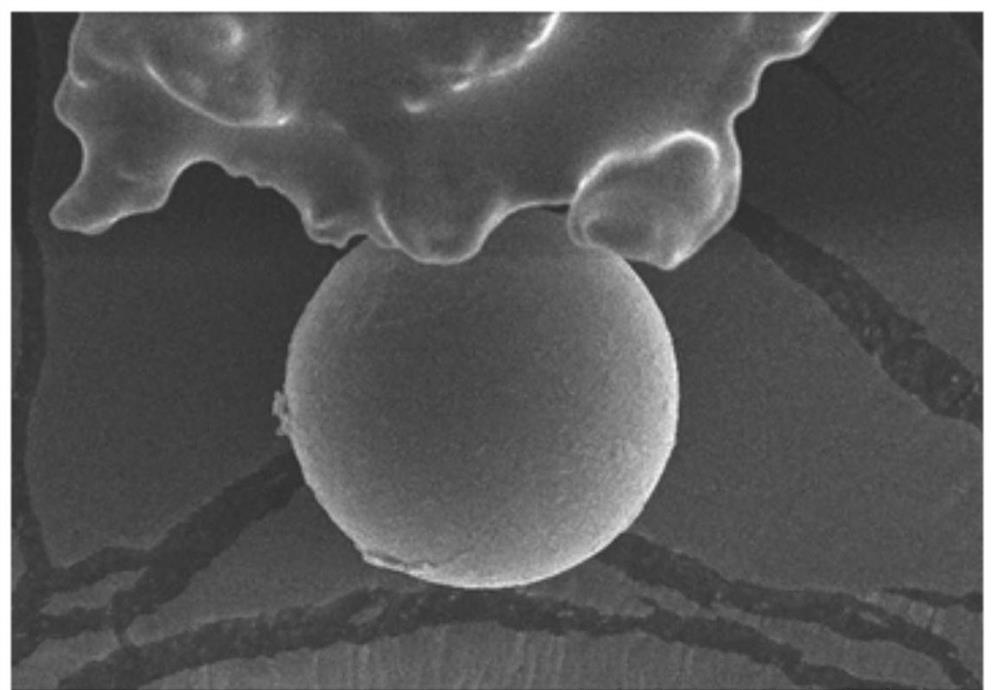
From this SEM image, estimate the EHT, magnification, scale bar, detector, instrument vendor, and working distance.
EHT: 10 kV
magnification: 1 K X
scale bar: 50000 nm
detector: SE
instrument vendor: Hitachi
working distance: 12 mm